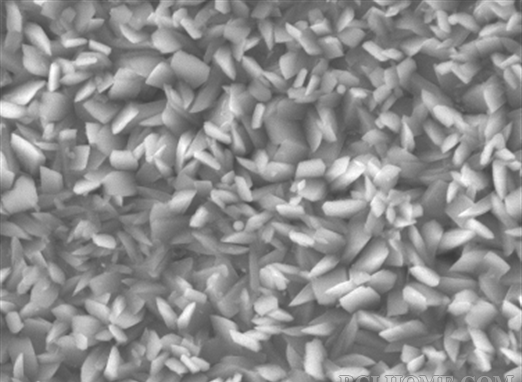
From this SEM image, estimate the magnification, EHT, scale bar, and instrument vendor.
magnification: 2 K X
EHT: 20 kV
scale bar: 5000 nm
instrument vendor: JEOL